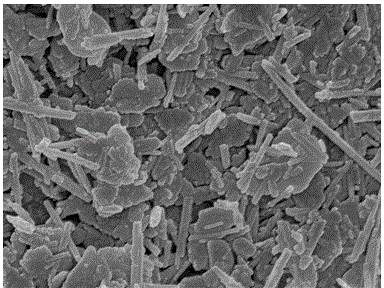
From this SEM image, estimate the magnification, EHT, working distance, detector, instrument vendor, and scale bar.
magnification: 40 K X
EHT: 5 kV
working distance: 7.9 mm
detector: SEI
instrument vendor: JEOL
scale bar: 100 nm